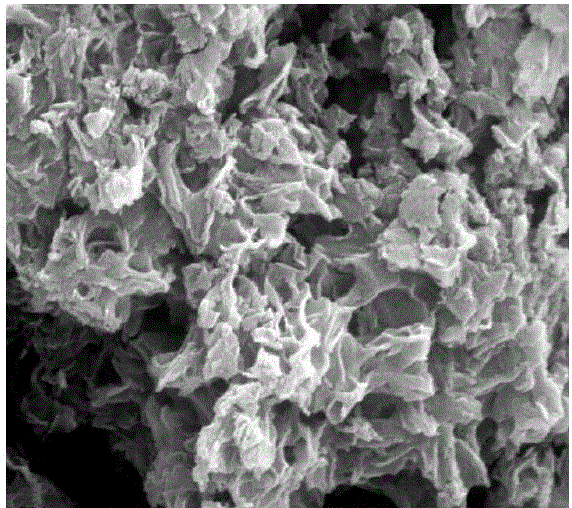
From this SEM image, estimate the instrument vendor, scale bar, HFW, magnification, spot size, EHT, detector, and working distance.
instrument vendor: FEI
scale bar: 500 nm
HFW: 2.98 µm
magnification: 50 K X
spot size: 3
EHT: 15 kV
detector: ETD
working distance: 9.9 mm